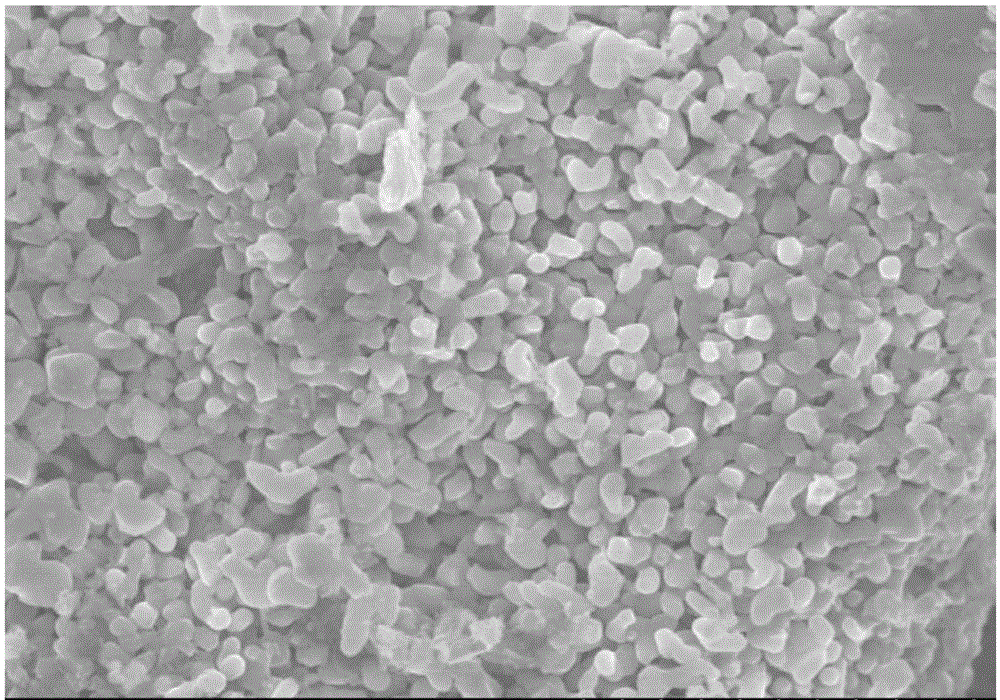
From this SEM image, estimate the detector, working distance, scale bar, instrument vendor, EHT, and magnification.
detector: SE(M)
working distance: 8.6 mm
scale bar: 5000 nm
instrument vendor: Hitachi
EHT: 10 kV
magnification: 10 K X